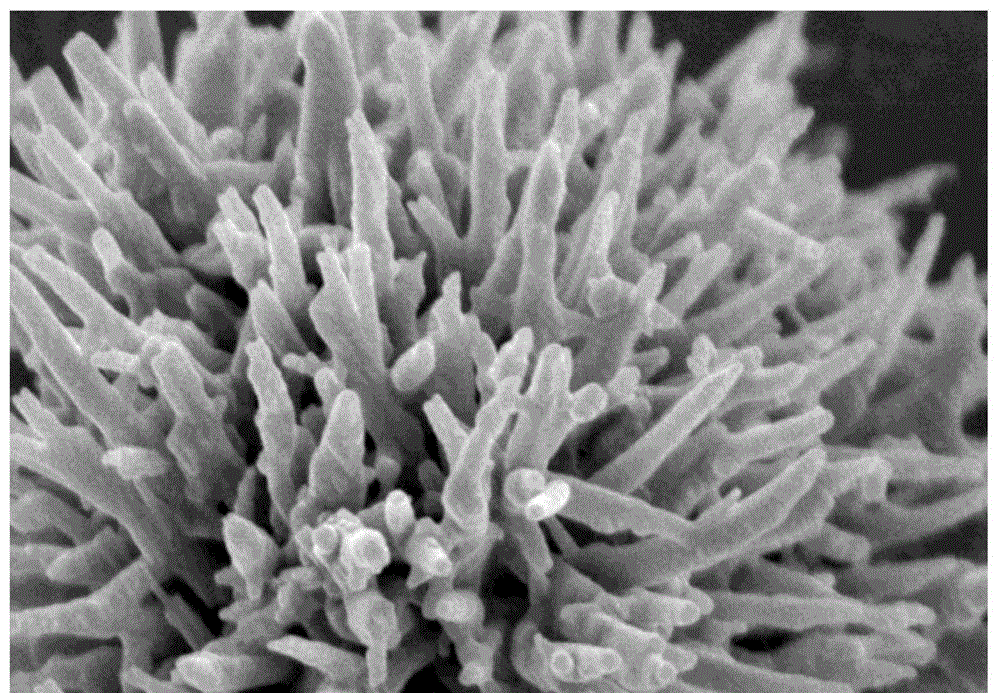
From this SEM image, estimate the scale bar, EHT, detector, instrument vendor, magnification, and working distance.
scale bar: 500 nm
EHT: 3 kV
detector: SE(M)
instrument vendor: Hitachi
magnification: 60 K X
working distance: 9.4 mm